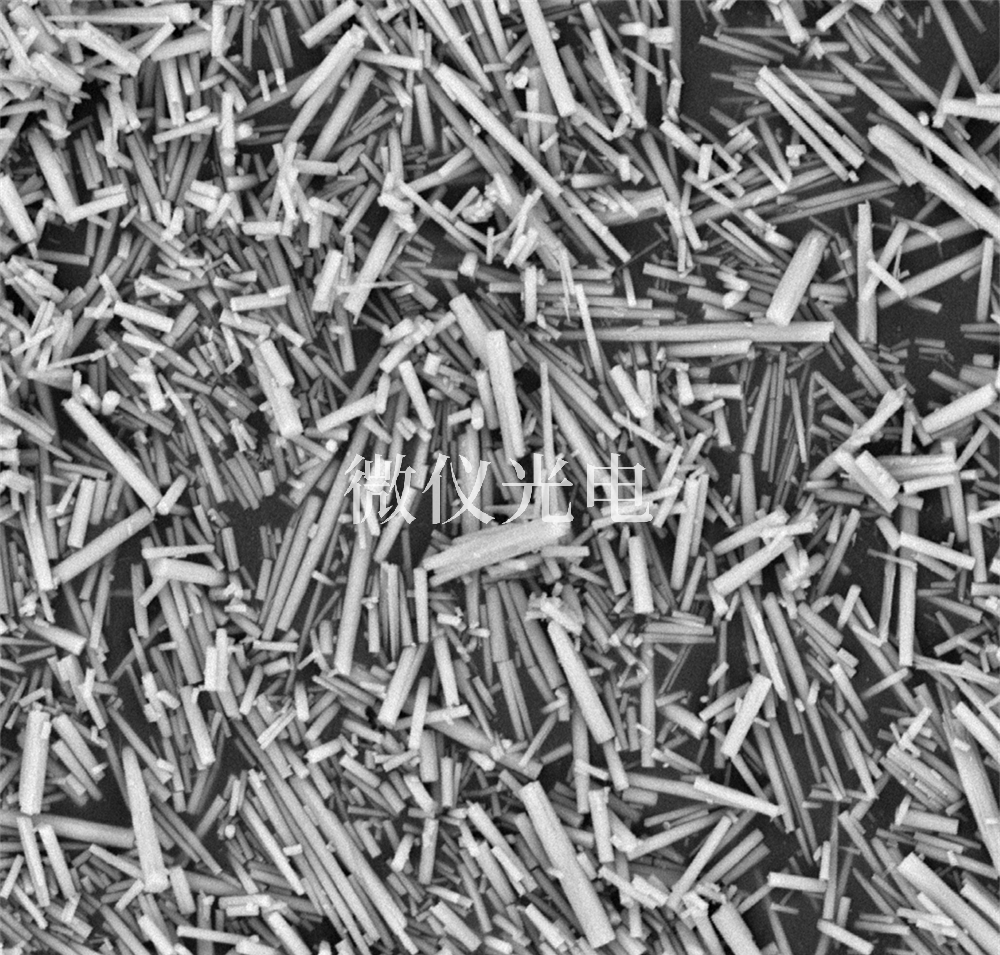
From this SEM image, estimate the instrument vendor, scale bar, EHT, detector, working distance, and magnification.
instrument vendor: other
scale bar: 5000 nm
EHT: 15 kV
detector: BSE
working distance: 5.8 mm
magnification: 10 K X